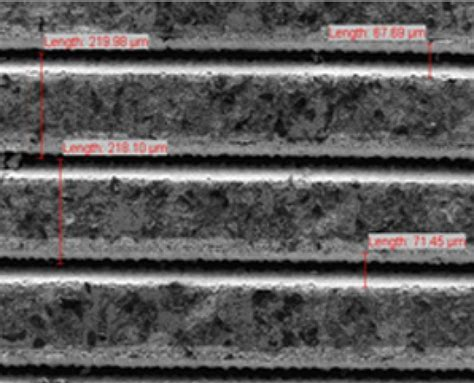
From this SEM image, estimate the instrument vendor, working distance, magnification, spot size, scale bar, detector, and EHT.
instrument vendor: FEI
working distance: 10.2 mm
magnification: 0.25 K X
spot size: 3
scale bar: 200000 nm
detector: SE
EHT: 10 kV